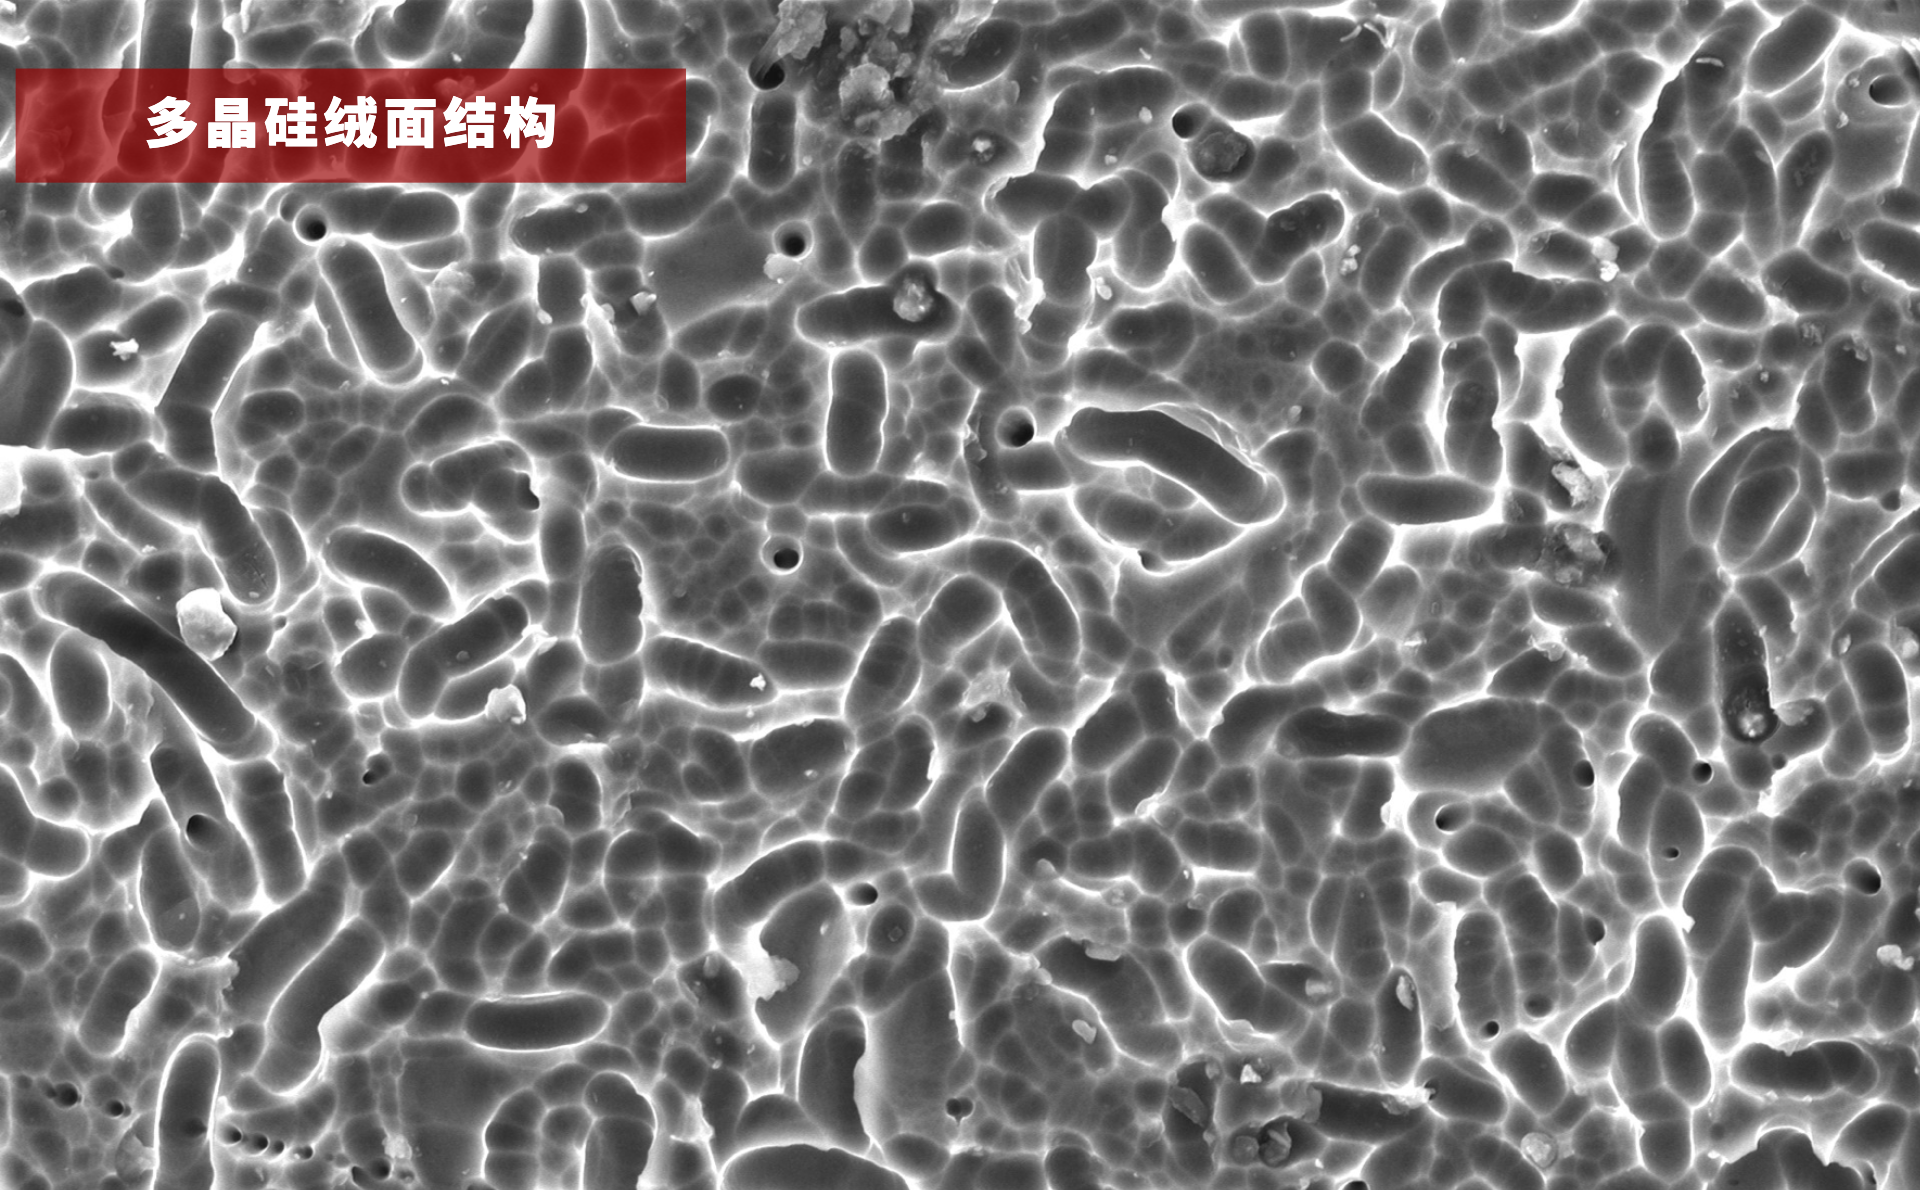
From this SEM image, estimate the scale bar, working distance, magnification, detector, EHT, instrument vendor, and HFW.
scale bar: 30000 nm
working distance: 10.893 mm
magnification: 1 K X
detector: SED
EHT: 15 kV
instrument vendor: other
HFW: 127 µm